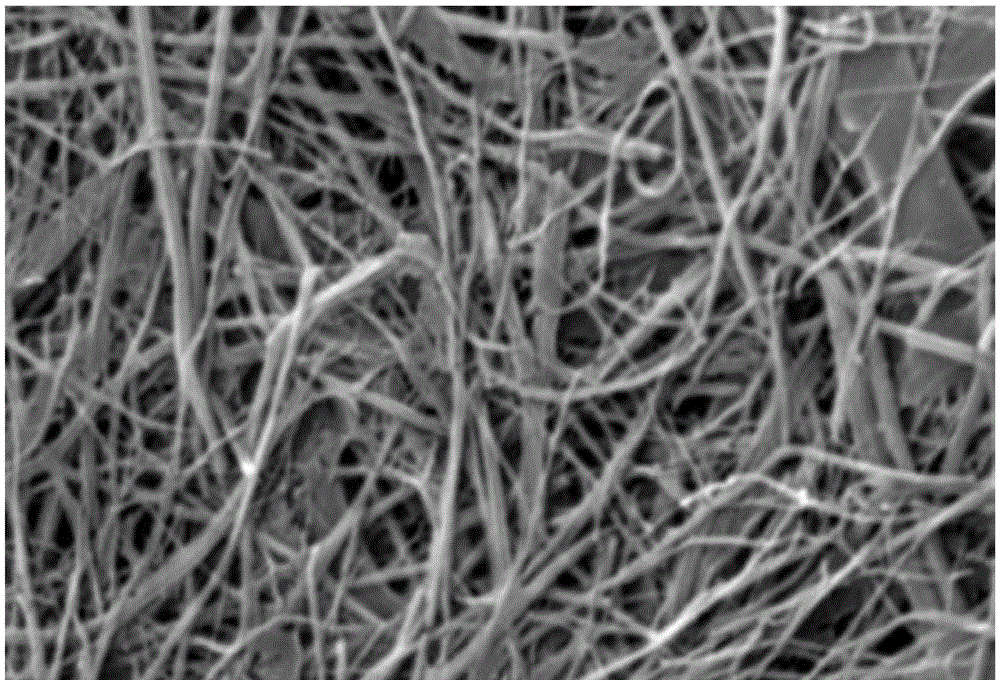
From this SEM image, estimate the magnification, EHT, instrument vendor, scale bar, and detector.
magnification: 5 K X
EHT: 20 kV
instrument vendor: JEOL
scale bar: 5000 nm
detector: SEI